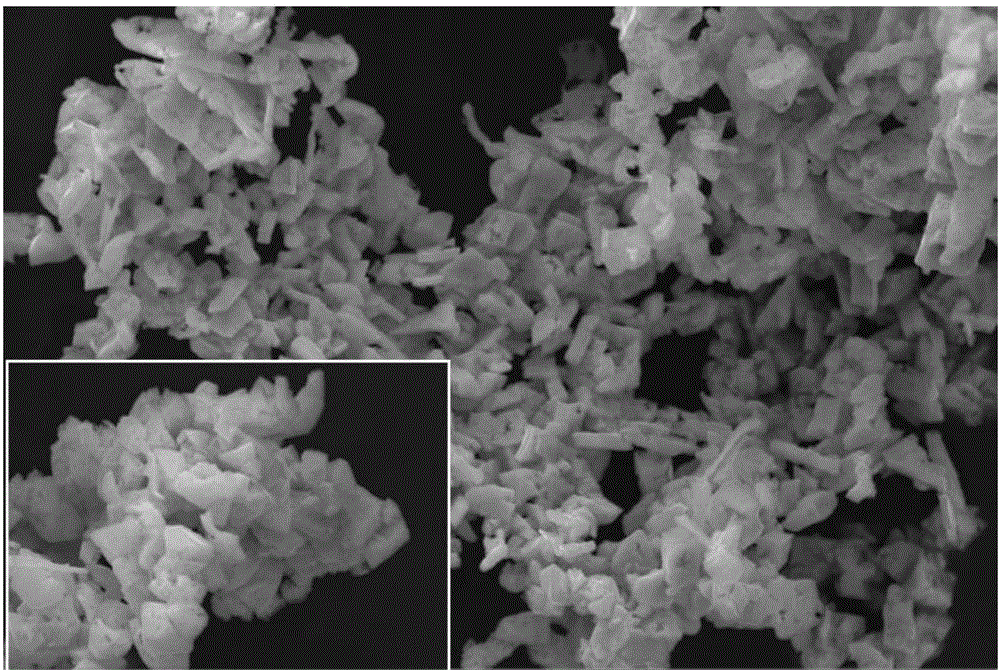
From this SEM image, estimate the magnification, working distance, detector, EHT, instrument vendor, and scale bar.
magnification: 10 K X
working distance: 8.5 mm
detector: ETD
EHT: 20 kV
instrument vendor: FEI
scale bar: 10000 nm